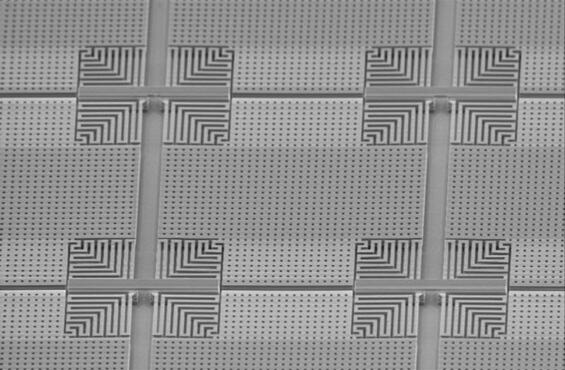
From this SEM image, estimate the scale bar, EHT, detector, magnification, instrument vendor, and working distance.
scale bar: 20000 nm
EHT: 3 kV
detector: SE2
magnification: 1.2 K X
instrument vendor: Zeiss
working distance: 13 mm